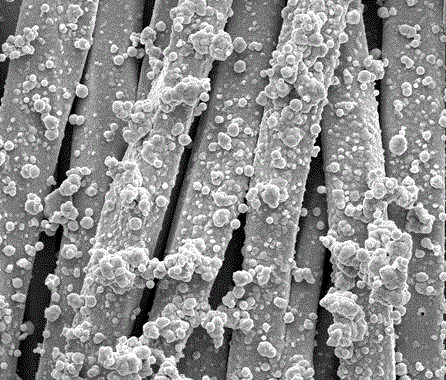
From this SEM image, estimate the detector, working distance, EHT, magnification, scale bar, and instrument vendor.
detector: SE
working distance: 11.7 mm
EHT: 20 kV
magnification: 3 K X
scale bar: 40000 nm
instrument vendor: FEI